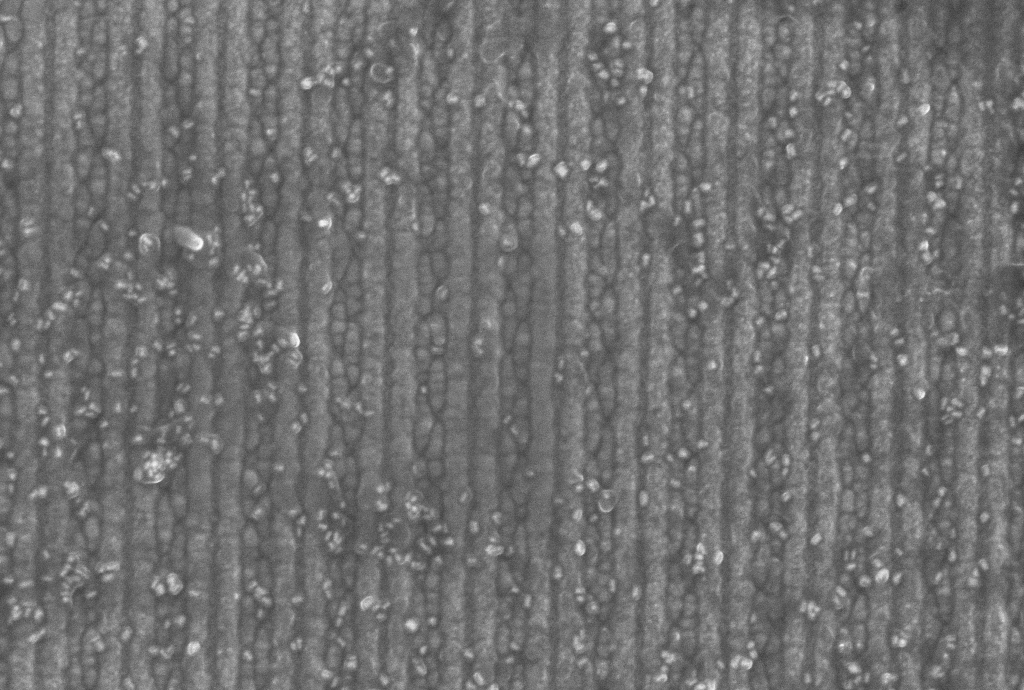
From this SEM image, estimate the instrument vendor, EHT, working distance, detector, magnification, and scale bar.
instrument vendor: Zeiss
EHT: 10 kV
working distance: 5.3 mm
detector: InLens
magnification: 100 K X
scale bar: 200 nm